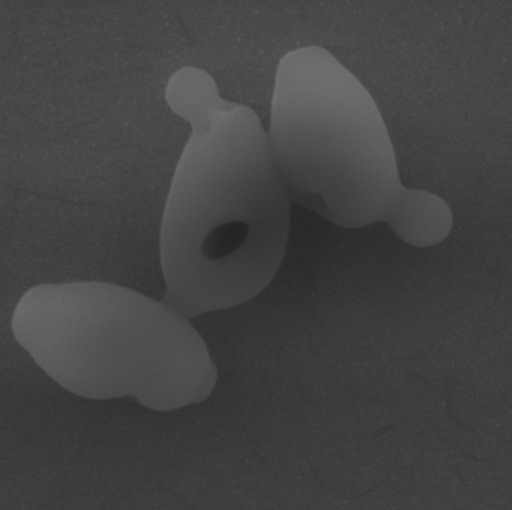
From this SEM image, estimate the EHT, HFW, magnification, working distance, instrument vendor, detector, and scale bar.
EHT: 10 kV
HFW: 6.82 µm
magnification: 20.3 K X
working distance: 5.07 mm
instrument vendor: Tescan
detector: SE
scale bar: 2000 nm